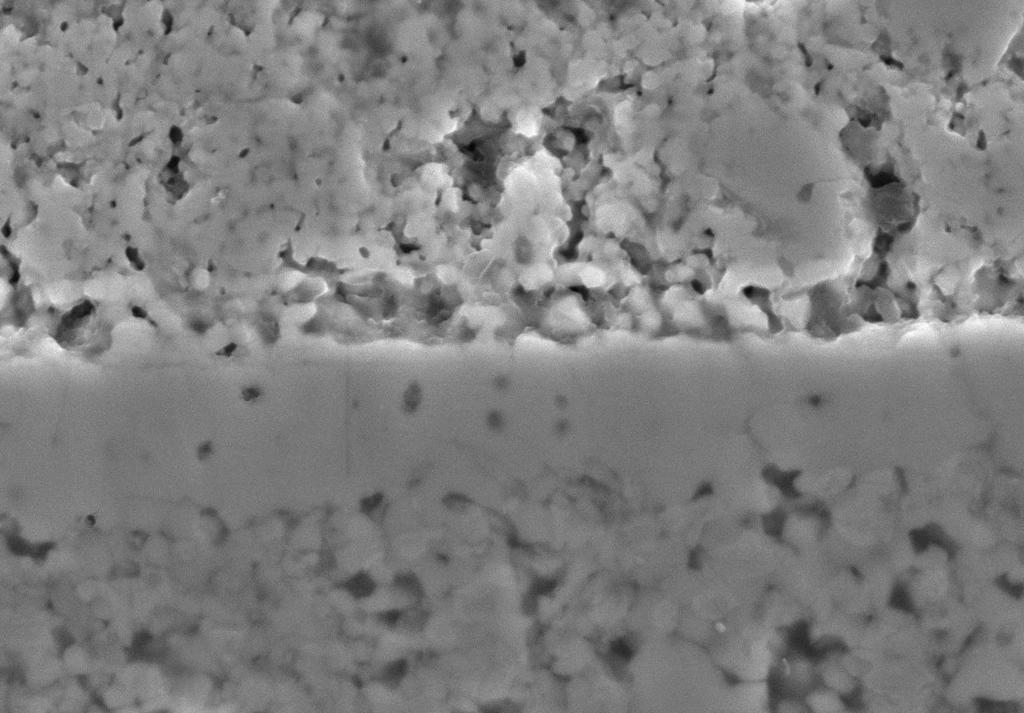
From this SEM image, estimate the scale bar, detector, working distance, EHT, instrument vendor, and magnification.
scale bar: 10000 nm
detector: SE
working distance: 10.2 mm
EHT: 20 kV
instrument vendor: Hitachi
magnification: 5 K X